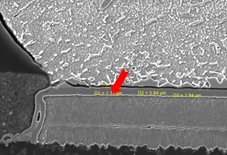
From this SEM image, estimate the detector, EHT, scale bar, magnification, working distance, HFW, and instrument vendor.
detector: SE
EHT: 20 kV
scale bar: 20000 nm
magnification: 2.2 K X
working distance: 11.94 mm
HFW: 126 µm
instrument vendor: Tescan